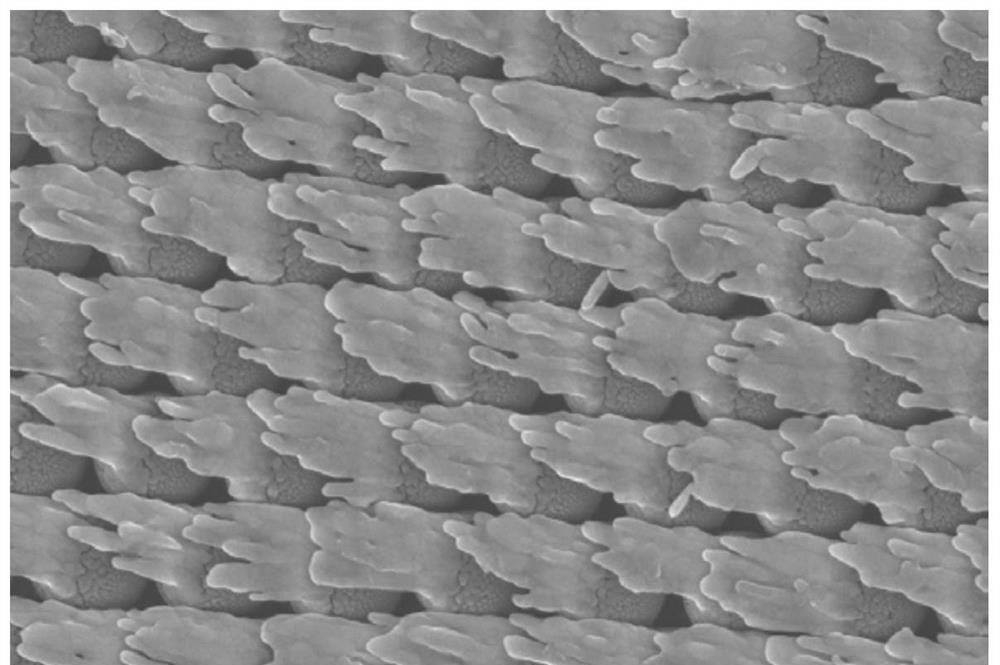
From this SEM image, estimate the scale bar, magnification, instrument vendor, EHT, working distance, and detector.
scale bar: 200 nm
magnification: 30 K X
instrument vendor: Zeiss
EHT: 15 kV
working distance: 6.8 mm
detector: InlensDuo